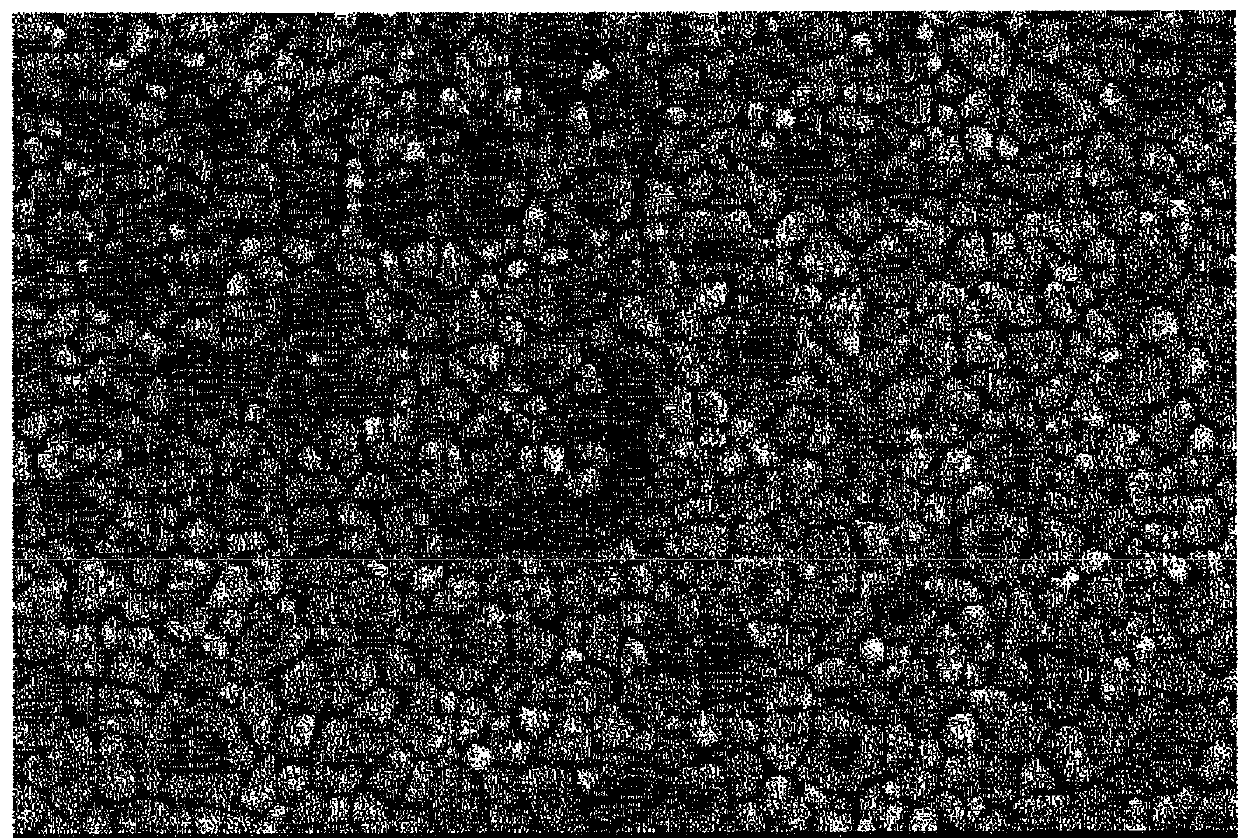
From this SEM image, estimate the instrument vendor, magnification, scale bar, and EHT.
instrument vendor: JEOL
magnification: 5 K X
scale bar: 5000 nm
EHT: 15 kV